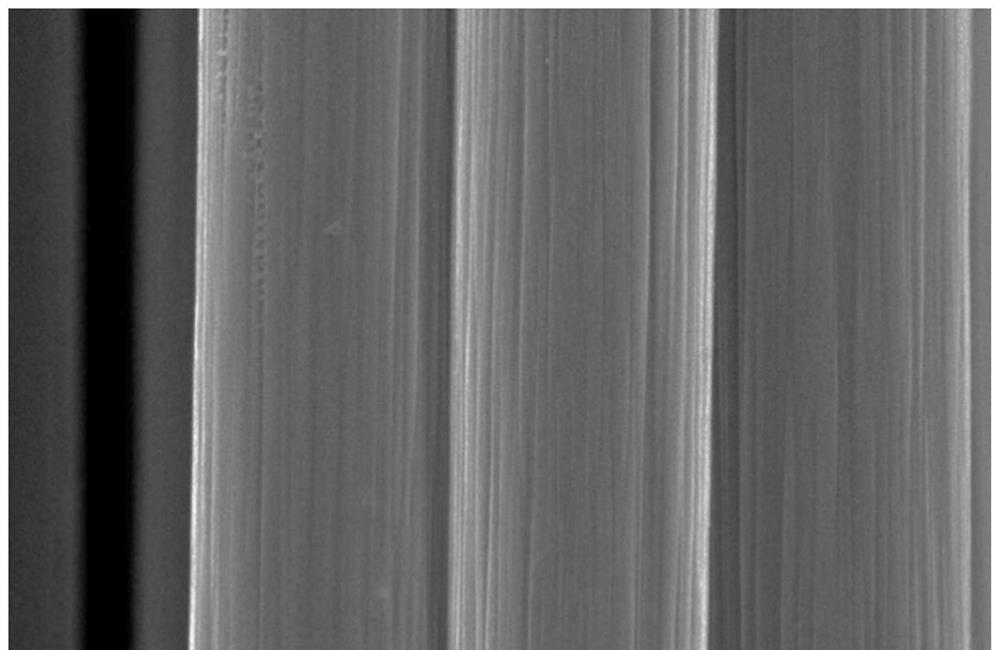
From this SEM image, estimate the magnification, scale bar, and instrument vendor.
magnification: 5 K X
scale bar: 5000 nm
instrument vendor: JEOL